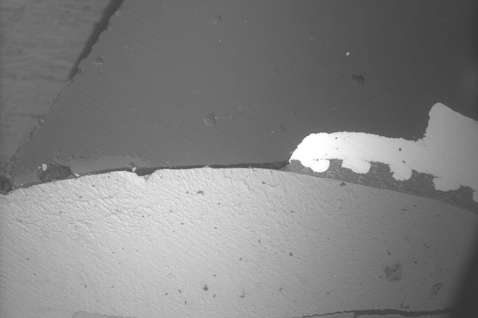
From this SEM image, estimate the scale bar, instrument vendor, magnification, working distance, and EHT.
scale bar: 600000 nm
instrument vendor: Tescan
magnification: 0.093 K X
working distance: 20.6 mm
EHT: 15 kV